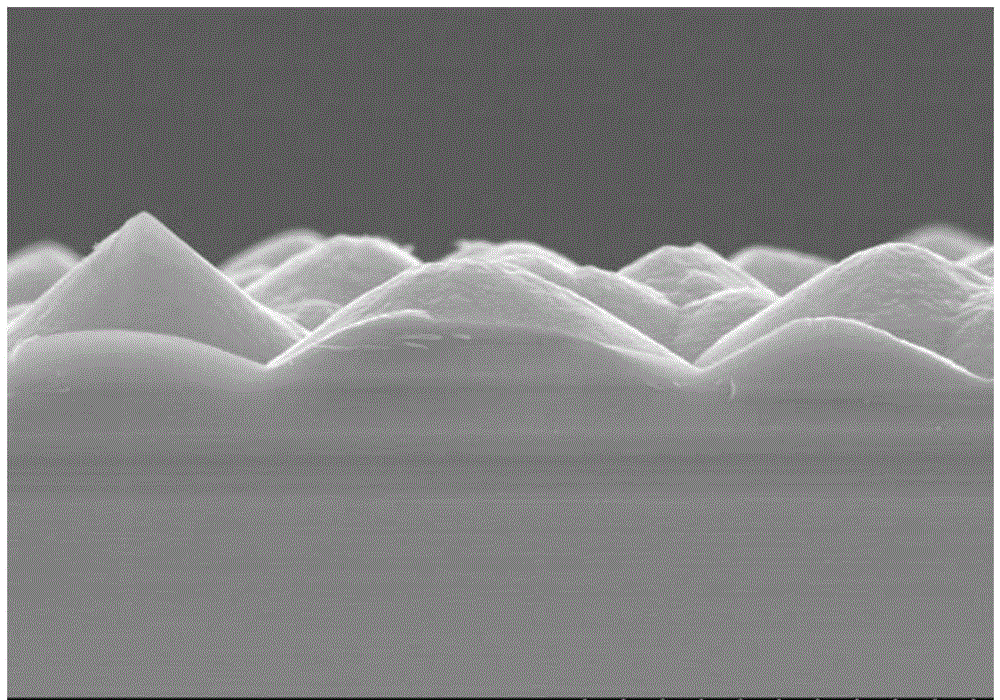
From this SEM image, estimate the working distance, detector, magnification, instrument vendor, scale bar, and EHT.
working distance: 9.8 mm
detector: SE(M)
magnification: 10 K X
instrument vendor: Hitachi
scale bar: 5000 nm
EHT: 10 kV